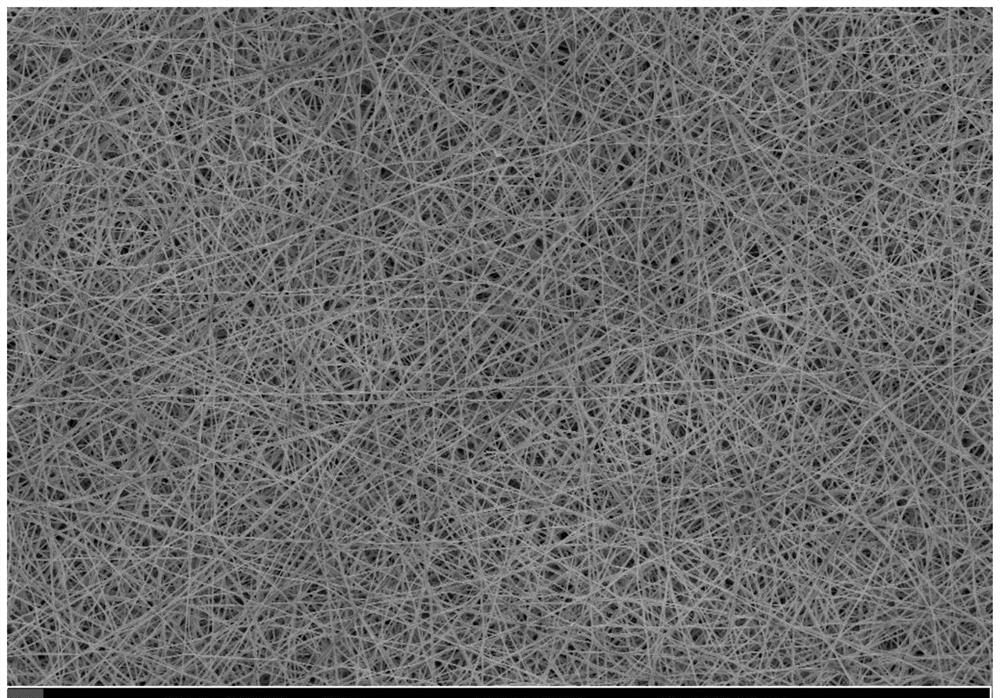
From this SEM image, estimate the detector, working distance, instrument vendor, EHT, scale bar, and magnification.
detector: InLens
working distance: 9.3 mm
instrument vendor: Zeiss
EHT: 5 kV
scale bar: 10000 nm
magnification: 1 K X